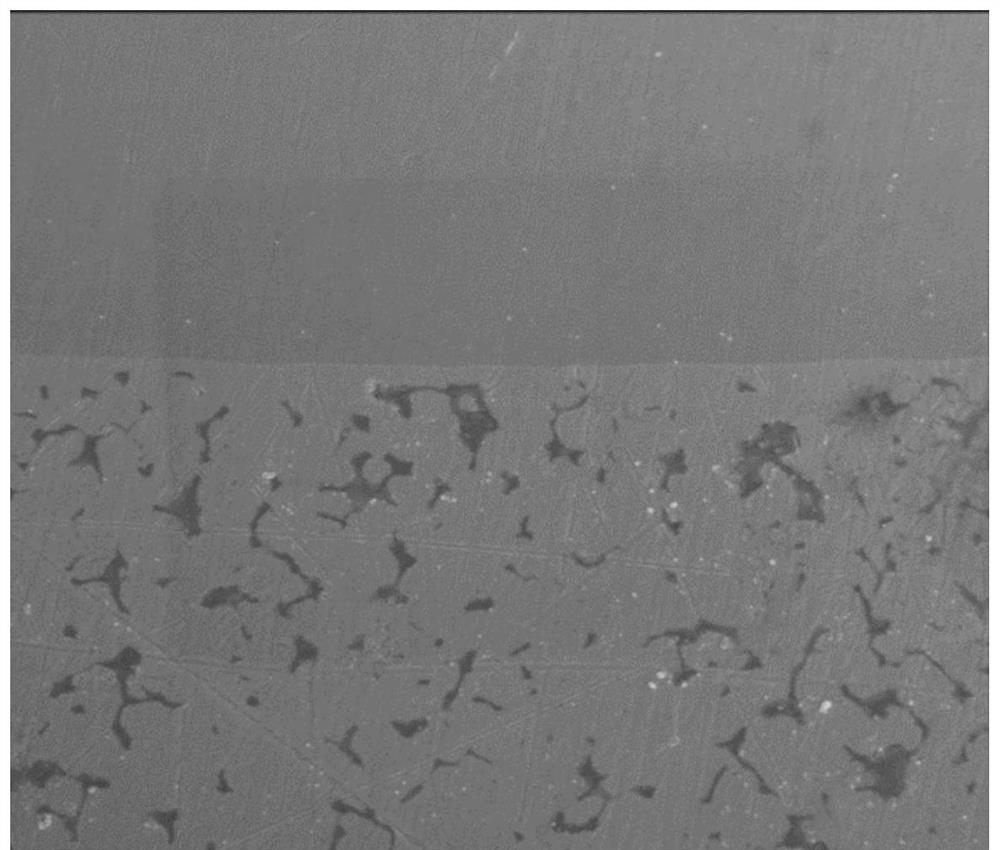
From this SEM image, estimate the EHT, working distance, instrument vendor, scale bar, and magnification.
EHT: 5 kV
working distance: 4.6 mm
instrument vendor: FEI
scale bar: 5000 nm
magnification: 10 K X